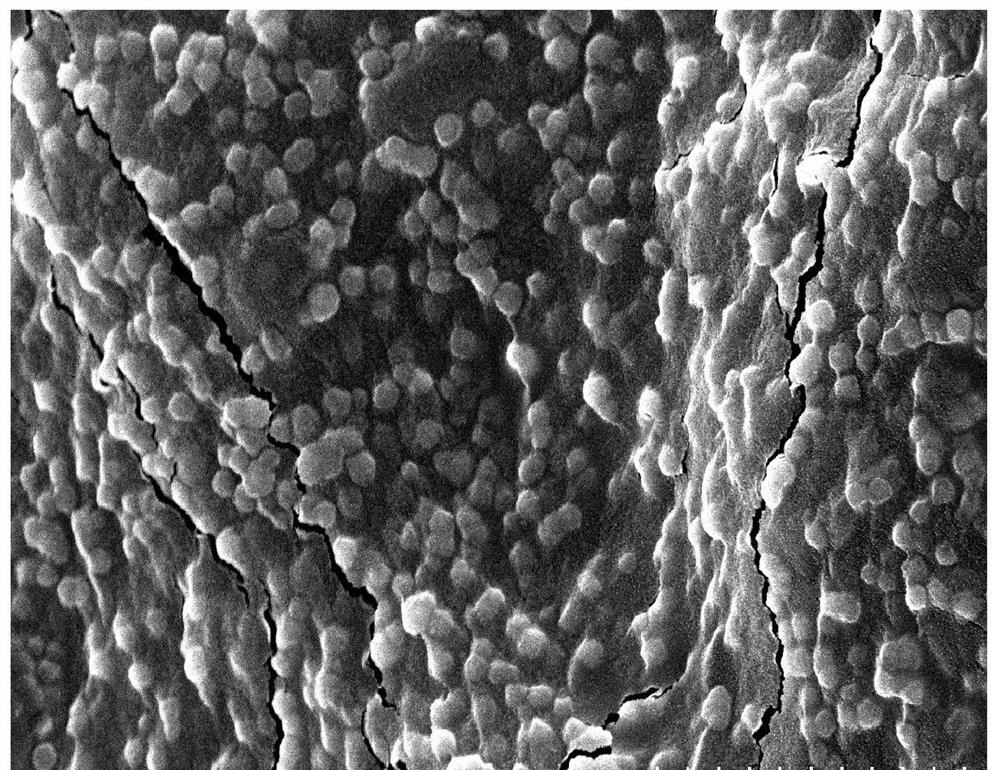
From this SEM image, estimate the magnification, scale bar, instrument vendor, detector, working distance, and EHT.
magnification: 20 K X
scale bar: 2000 nm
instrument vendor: Hitachi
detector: SE(UL)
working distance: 10.5 mm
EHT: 5 kV